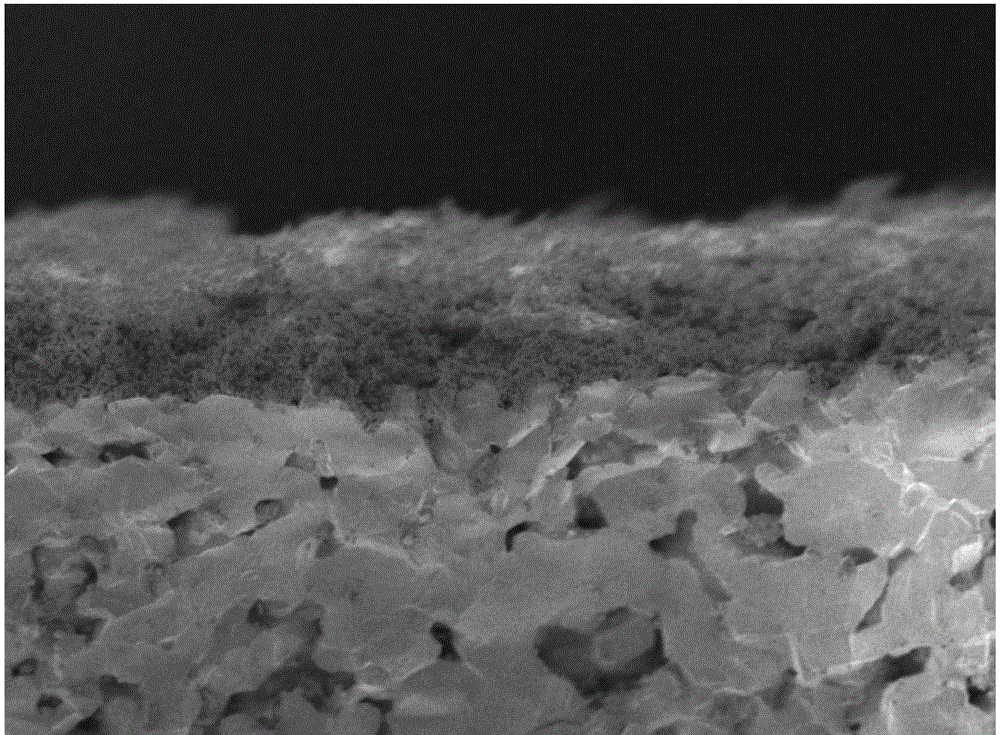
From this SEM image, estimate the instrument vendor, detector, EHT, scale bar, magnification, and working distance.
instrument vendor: JEOL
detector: SEI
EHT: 1 kV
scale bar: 10000 nm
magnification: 2 K X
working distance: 7.8 mm